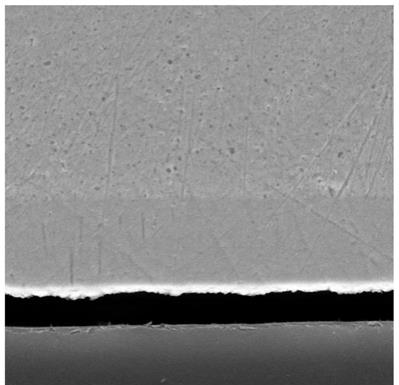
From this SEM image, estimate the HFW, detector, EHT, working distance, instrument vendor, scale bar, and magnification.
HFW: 25.4 µm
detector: SE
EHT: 15 kV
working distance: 9.98 mm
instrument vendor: Tescan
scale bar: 5000 nm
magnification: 10 K X